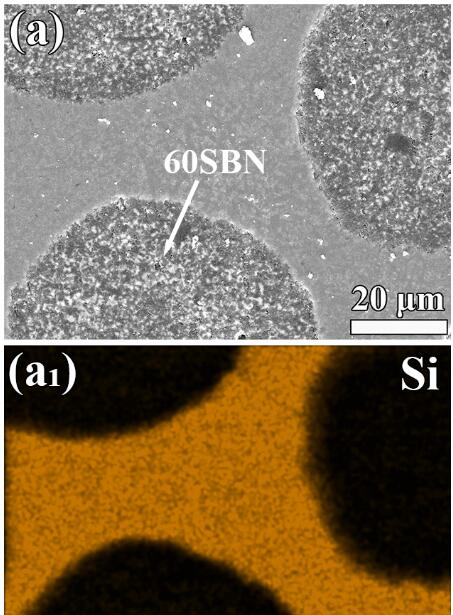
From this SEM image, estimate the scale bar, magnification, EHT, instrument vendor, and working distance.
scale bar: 20000 nm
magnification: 3.001 K X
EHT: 15 kV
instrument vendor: other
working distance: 20 mm